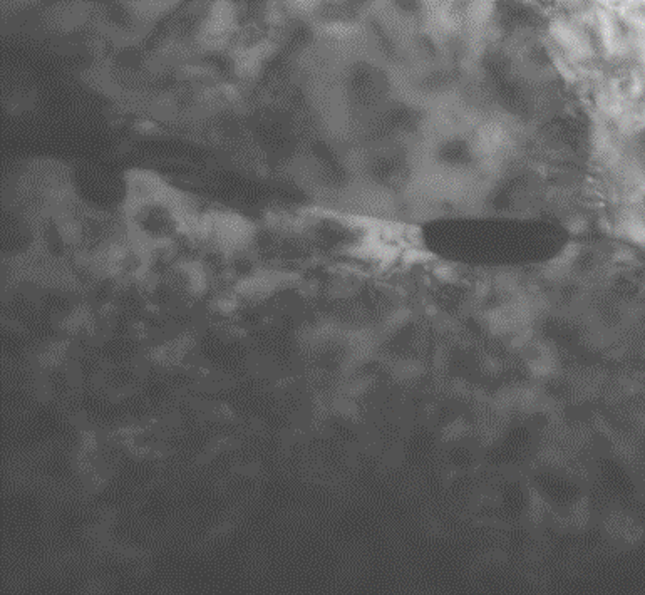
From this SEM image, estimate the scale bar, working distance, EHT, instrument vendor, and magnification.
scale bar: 20 nm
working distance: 4.5 mm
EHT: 30 kV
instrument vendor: Zeiss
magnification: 150 K X